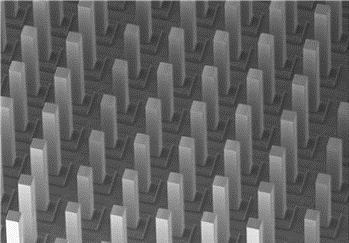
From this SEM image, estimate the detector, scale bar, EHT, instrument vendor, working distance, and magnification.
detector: LM(UL)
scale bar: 1000000 nm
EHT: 3 kV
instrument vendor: Hitachi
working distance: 13.6 mm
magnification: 0.03 K X